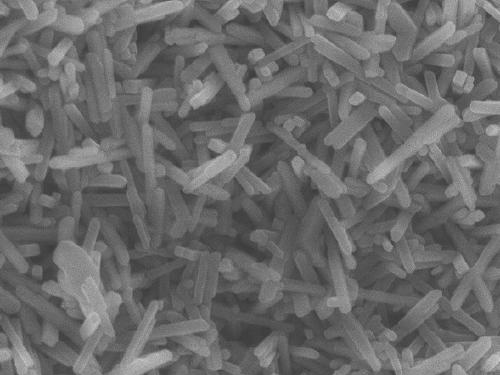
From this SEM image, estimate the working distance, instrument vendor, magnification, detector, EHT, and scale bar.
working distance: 6 mm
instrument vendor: JEOL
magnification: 60 K X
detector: SEI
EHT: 5 kV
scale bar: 100 nm